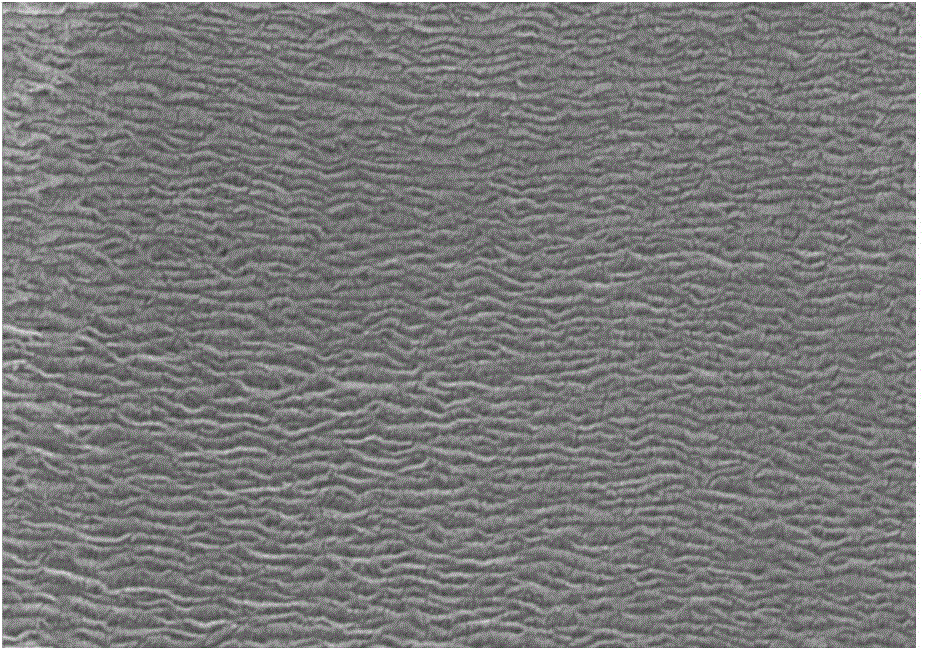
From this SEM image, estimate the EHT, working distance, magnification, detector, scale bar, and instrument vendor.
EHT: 20 kV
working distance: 10.4 mm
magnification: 50 K X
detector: InLens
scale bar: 100 nm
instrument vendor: Zeiss